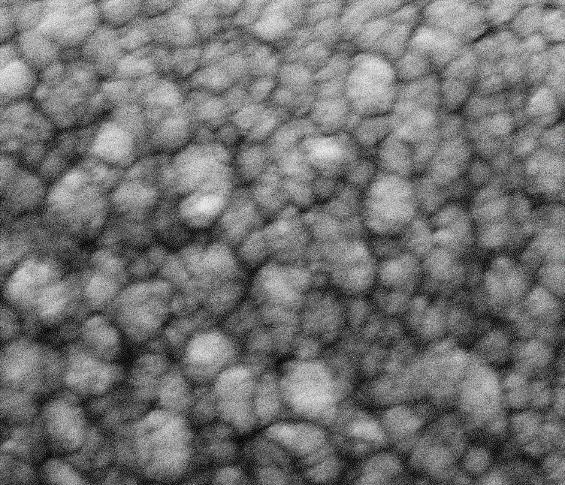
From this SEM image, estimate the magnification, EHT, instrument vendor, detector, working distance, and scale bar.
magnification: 200 K X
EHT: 10 kV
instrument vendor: FEI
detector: ETD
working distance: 7.3 mm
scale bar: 500 nm